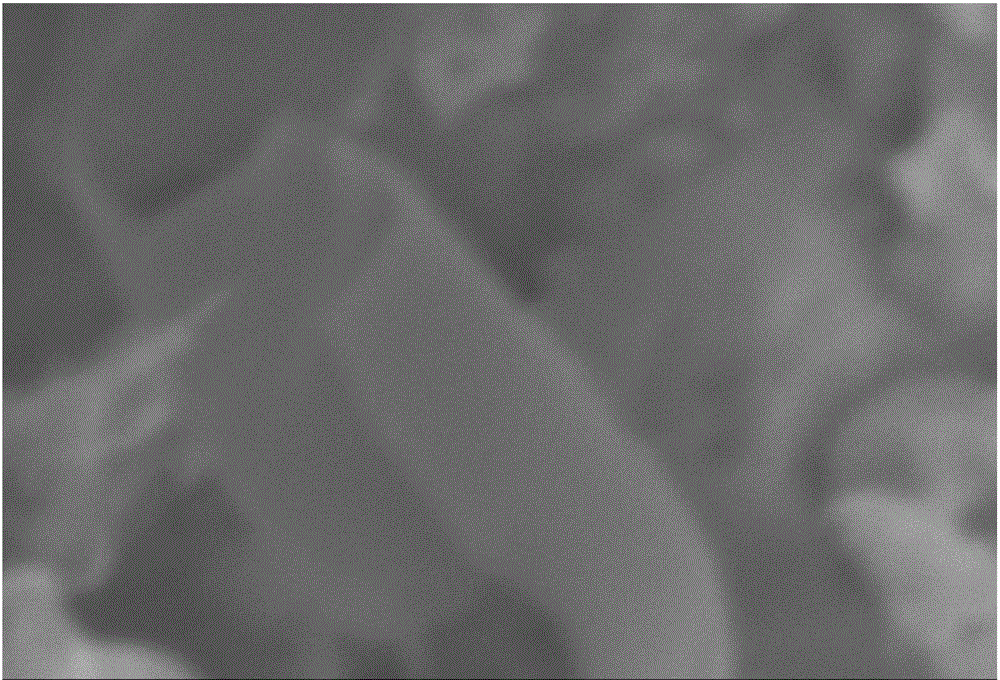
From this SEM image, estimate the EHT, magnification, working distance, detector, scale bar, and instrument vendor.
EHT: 15 kV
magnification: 20 K X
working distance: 23 mm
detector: SEI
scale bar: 1000 nm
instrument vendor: JEOL